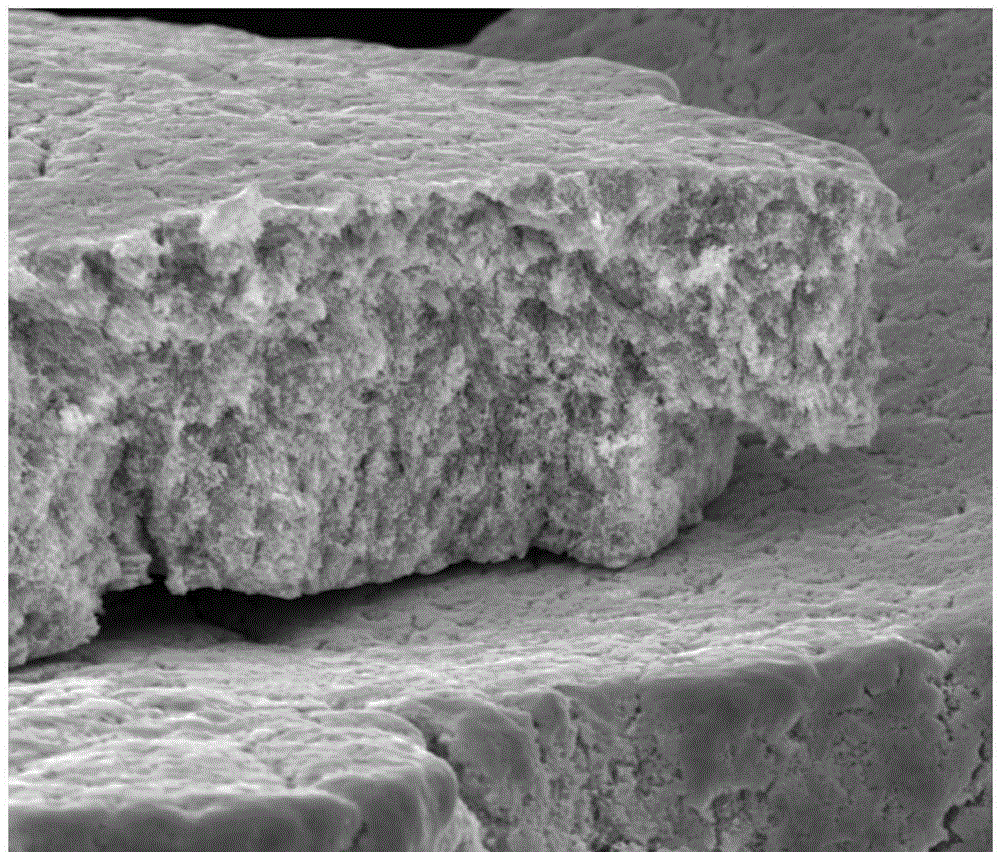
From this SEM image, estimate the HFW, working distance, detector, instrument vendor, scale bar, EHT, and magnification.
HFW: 42.3 µm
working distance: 11.1 mm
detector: ETD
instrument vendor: FEI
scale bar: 20000 nm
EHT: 20 kV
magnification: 3 K X